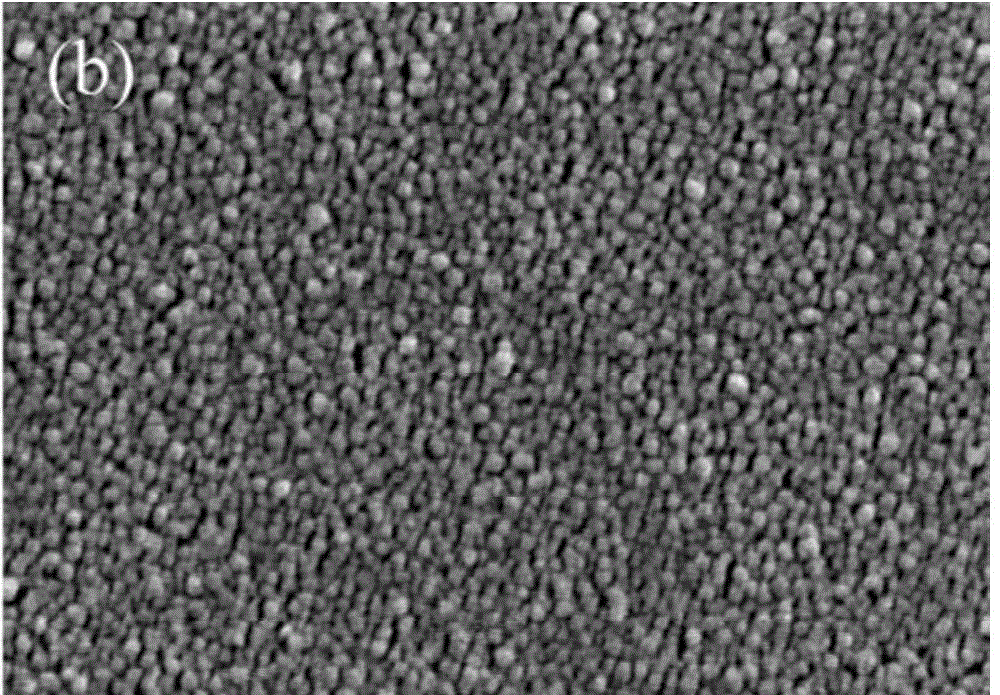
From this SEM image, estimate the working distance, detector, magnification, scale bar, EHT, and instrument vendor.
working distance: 4 mm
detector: SE(M)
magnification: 200 K X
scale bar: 200 nm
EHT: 5 kV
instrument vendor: Hitachi